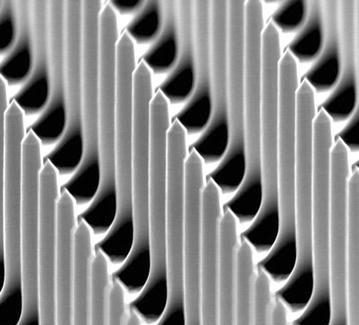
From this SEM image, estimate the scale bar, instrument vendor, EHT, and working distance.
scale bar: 5000 nm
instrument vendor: FEI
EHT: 20 kV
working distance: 7.1 mm